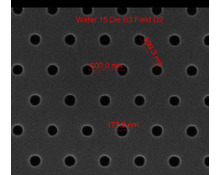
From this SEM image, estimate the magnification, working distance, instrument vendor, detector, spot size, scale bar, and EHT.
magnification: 75 K X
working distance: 10.3 mm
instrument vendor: FEI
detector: ETD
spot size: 1.5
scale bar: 1000 nm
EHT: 10 kV